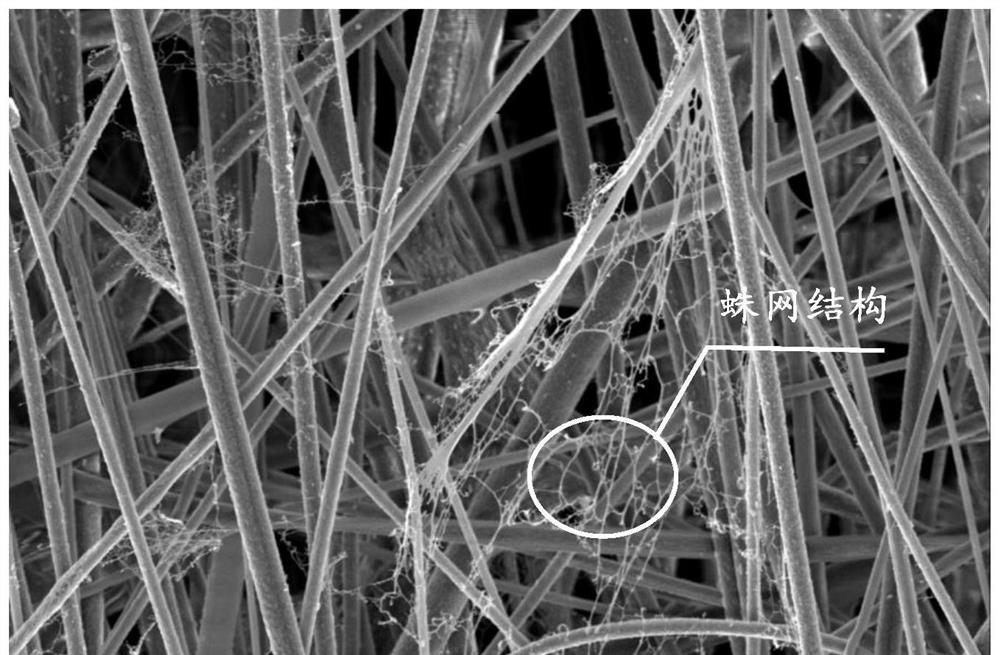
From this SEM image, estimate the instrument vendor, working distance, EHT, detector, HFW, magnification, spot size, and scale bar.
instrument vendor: FEI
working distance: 9.5 mm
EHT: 5 kV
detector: ETD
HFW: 41.4 µm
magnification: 10 K X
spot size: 8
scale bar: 10000 nm